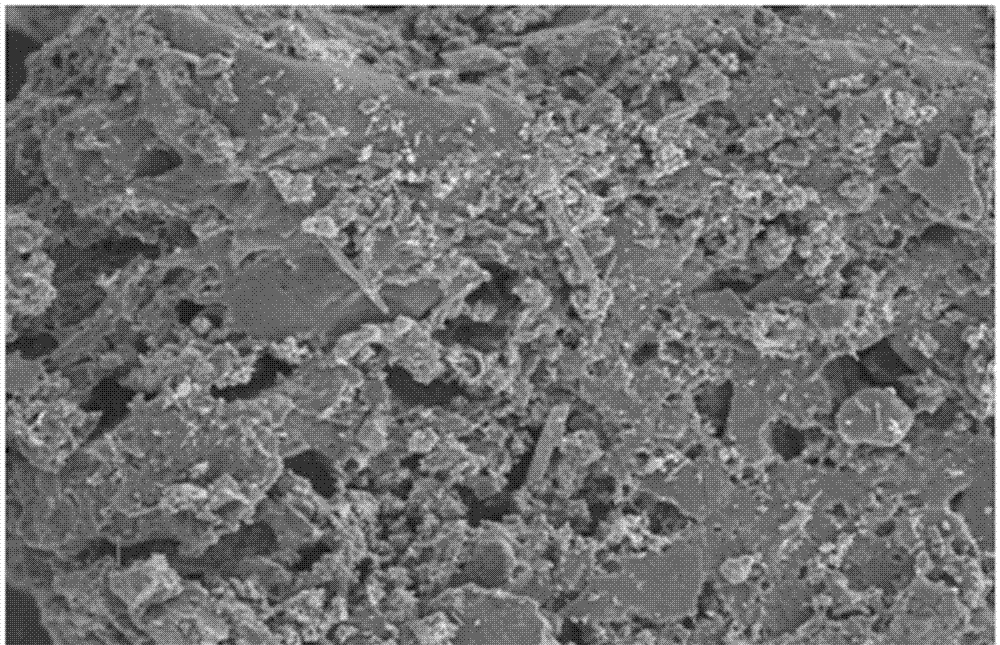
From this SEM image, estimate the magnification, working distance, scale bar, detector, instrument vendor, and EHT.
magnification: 2 K X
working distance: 7.8 mm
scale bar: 2000 nm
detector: SE2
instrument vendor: Zeiss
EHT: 3 kV